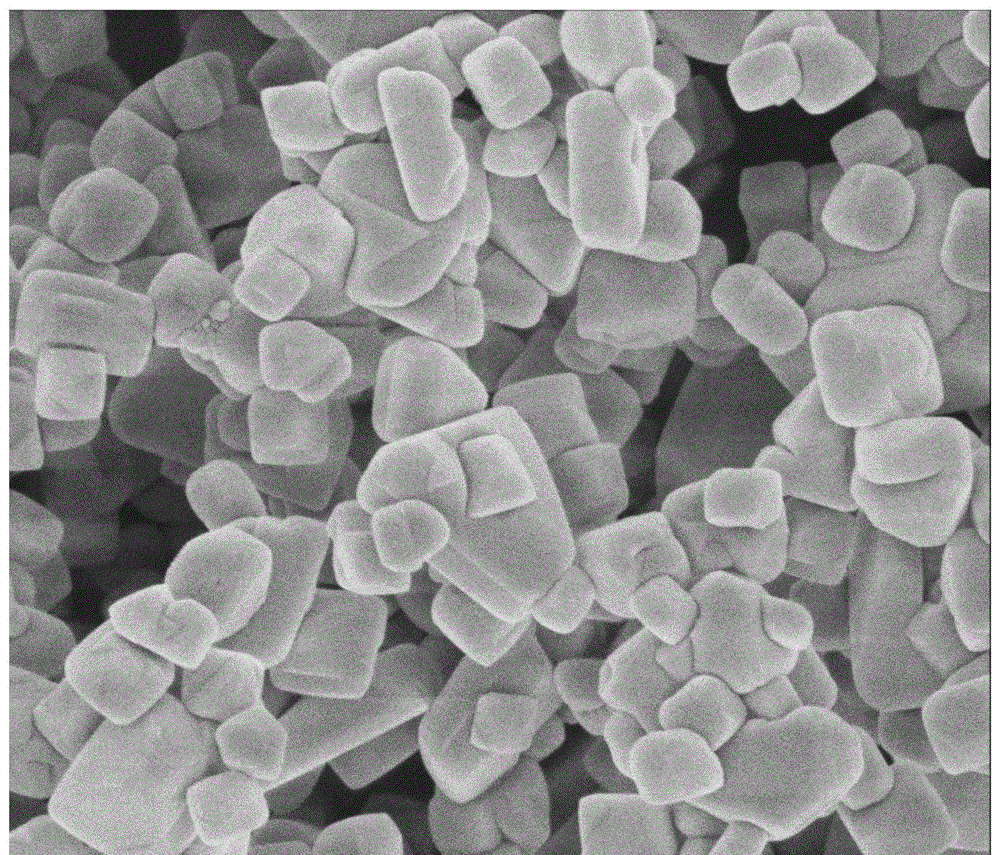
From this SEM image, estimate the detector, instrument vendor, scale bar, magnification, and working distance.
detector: TLD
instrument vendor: FEI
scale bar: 1000 nm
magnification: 40 K X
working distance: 4.5 mm